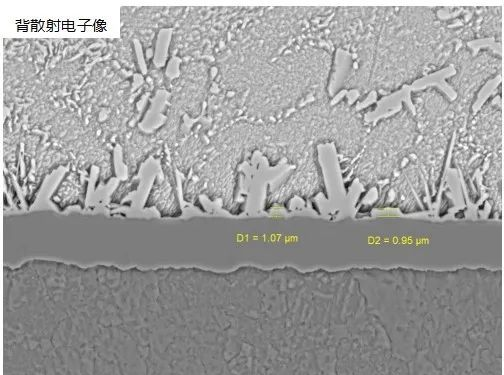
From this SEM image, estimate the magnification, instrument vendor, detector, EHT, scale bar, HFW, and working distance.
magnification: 4.82 K X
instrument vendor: Tescan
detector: BSE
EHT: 20 kV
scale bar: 10000 nm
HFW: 57.4 µm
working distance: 10.01 mm